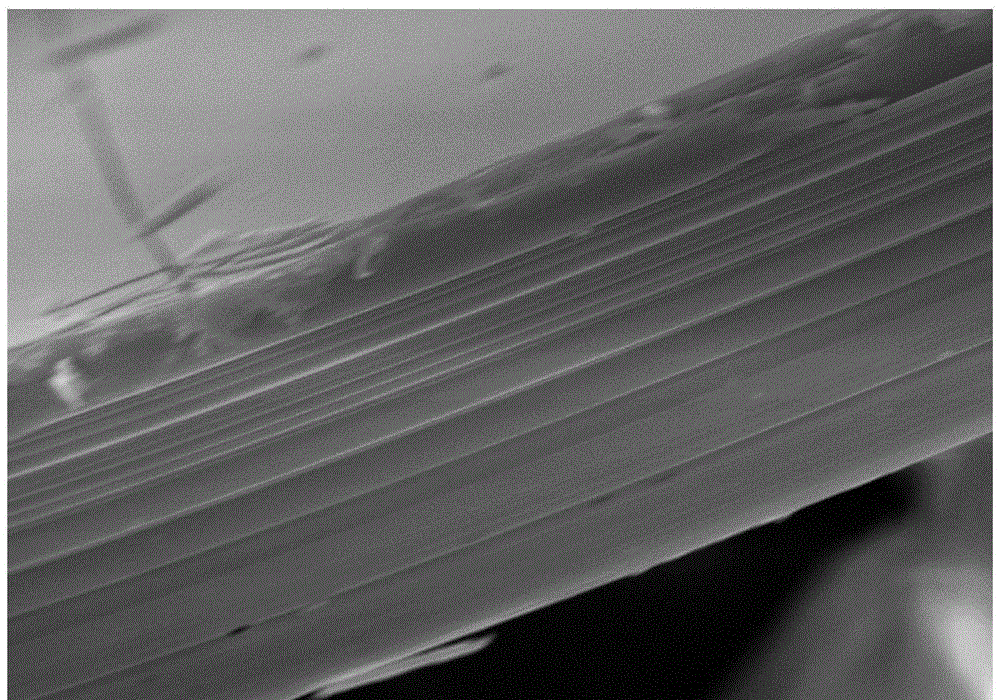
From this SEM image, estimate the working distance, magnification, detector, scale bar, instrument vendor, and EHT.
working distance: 4.9 mm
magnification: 10 K X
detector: InLens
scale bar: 1000 nm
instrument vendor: Zeiss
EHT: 10 kV